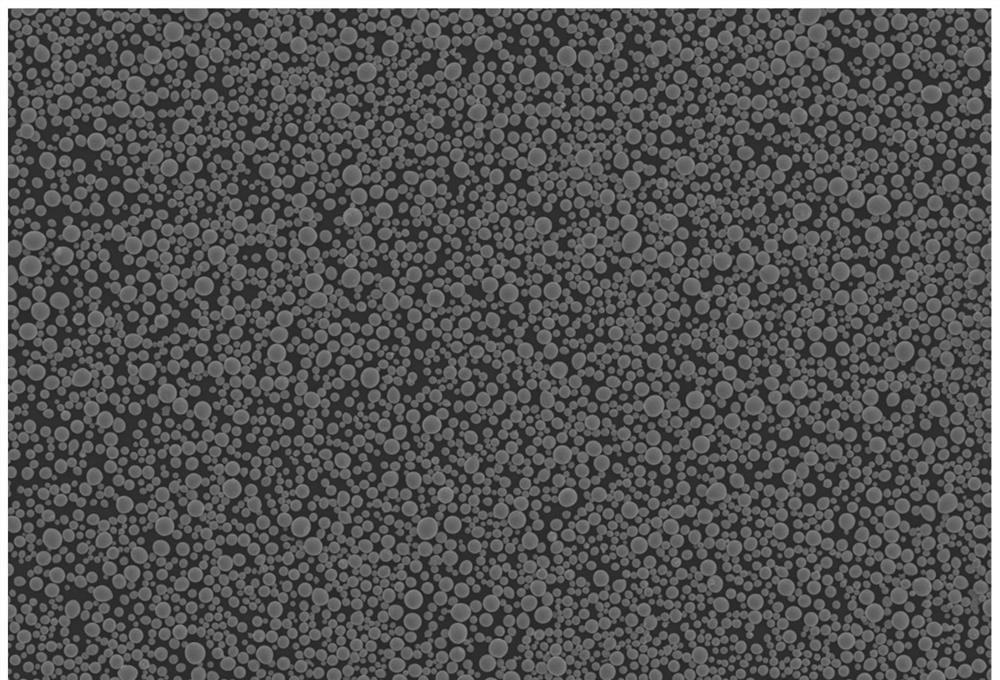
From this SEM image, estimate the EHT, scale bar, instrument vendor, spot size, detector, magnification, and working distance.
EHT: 30 kV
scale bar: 100000 nm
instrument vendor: JEOL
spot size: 32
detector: SEI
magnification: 0.1 K X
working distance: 11 mm